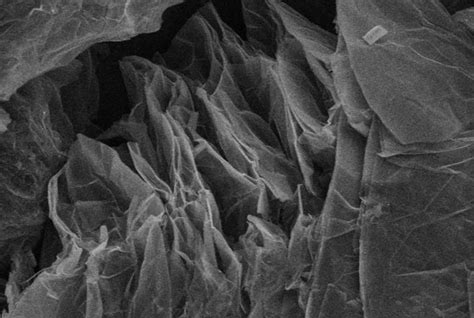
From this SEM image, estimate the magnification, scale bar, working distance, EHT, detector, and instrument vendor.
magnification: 1.2 K X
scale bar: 40000 nm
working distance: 8.6 mm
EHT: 10 kV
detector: SE(M)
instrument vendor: Hitachi